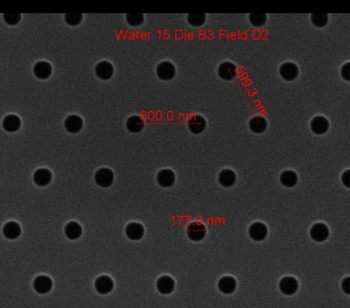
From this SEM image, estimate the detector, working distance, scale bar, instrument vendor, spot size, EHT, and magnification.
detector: ETD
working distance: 10.3 mm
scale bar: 1000 nm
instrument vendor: FEI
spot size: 1.5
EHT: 10 kV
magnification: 75 K X